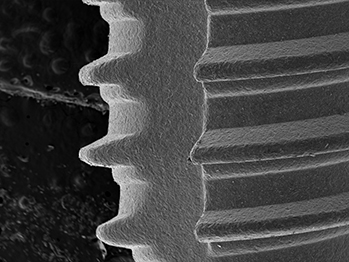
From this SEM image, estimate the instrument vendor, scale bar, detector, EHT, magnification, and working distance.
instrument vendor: other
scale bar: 1000000 nm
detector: SE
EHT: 15 kV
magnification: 0.056 K X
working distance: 40.8 mm